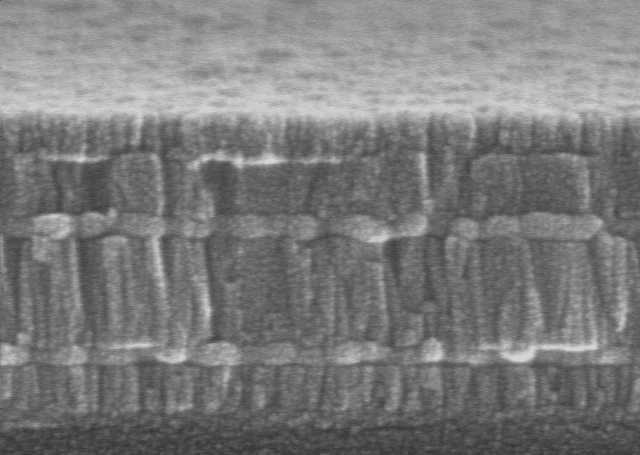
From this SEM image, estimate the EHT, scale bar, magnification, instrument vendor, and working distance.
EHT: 15 kV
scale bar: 200 nm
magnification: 200 K X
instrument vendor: JEOL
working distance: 5 mm